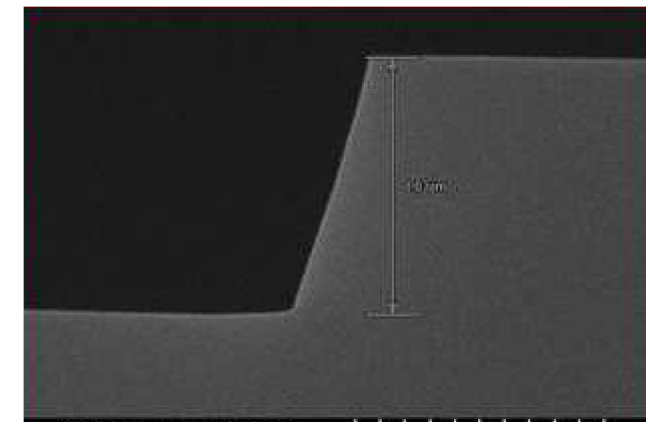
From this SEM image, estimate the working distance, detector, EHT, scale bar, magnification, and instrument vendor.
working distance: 9.8 mm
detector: SE(U)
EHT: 10 kV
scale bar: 1000 nm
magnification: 50 K X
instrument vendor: Hitachi